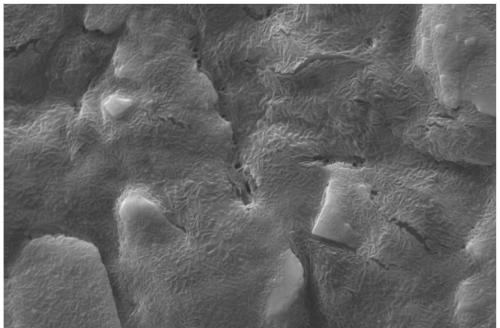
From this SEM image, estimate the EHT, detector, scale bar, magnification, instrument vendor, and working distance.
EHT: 20 kV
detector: SE1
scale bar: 2000 nm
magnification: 3 K X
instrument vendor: Zeiss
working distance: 8 mm